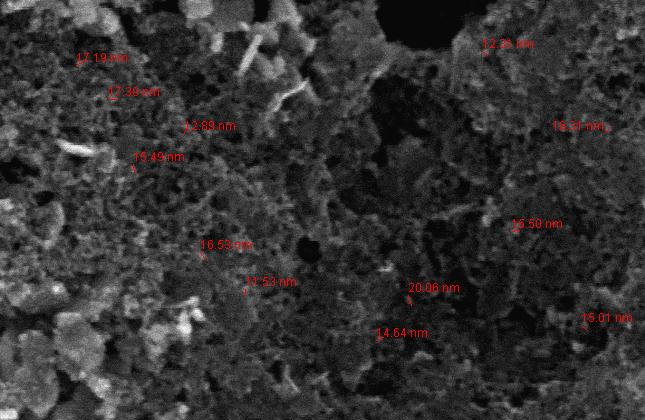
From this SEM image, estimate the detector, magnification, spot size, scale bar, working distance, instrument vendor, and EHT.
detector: TLD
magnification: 100 K X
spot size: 2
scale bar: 200 nm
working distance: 4.1 mm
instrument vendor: FEI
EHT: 7 kV